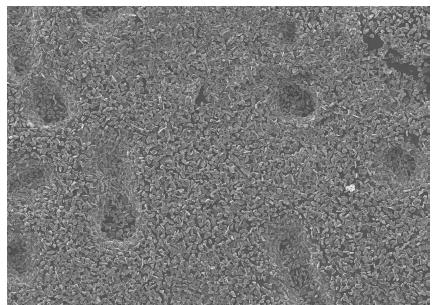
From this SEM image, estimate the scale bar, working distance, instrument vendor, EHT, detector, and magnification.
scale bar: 100000 nm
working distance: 19.5 mm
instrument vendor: JEOL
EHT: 15 kV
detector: SEI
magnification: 0.1 K X